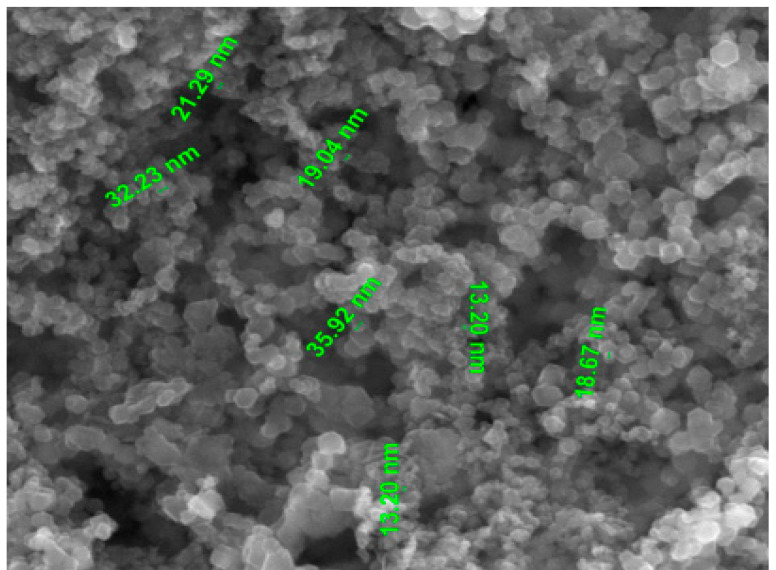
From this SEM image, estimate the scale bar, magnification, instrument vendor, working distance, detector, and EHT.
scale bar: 1000 nm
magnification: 100 K X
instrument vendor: FEI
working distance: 9.6 mm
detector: ETD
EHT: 30 kV